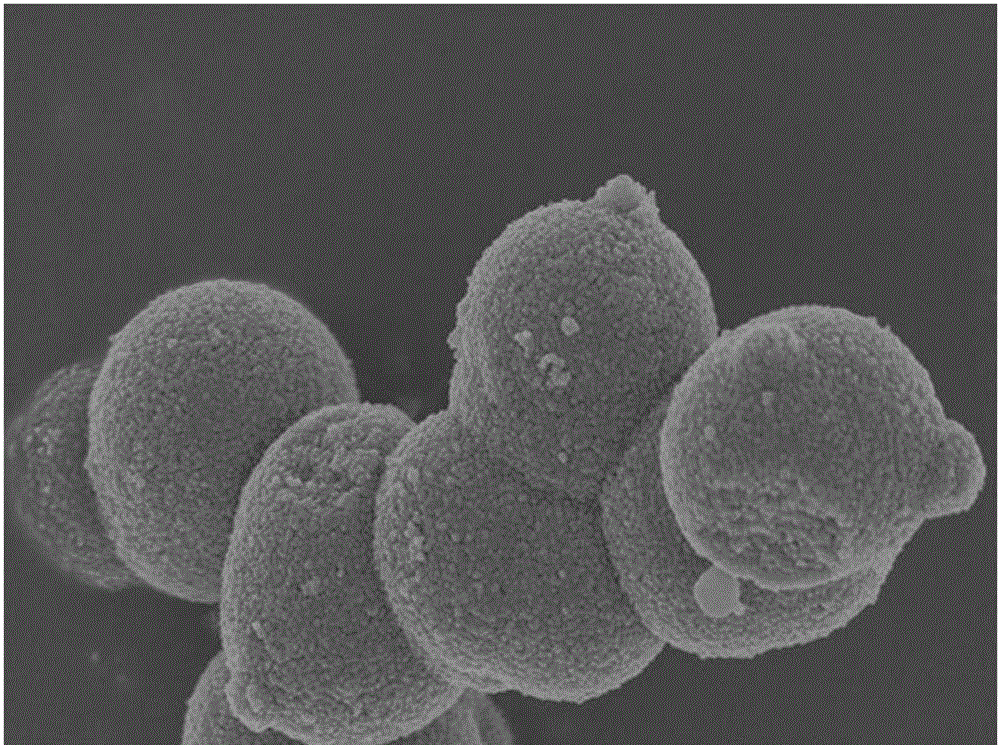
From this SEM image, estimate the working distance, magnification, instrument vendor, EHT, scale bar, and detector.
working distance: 9 mm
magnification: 25 K X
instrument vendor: Hitachi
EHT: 3 kV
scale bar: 2000 nm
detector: SE(U)